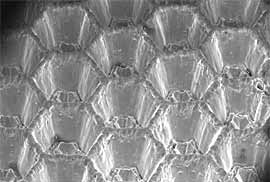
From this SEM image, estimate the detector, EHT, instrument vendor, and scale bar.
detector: QBSD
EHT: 25 kV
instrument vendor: Zeiss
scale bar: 1000000 nm